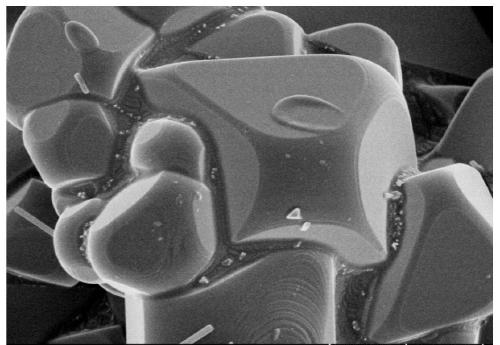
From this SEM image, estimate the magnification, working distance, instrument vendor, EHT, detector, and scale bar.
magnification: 20 K X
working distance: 3.8 mm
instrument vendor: Hitachi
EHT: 3 kV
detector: SE(TUL)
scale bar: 2000 nm